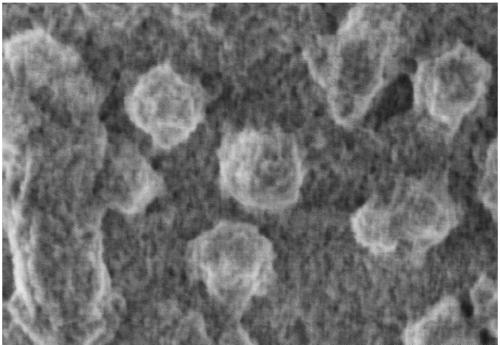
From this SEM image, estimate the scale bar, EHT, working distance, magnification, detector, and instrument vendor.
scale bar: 500 nm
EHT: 1 kV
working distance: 1.8 mm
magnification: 80 K X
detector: SE(U)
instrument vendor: Hitachi